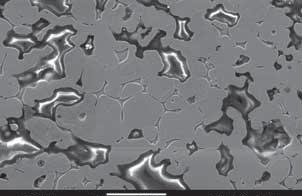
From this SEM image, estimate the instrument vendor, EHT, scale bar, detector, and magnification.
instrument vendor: JEOL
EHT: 20 kV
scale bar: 50000 nm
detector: SEI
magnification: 0.5 K X